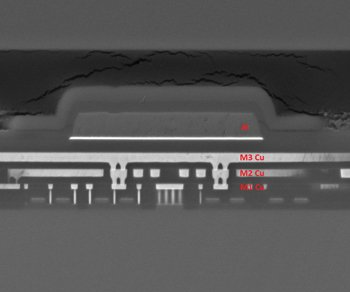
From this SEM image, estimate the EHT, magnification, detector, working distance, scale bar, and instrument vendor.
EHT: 10 kV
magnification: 20 K X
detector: BSED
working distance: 9.7 mm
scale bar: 5000 nm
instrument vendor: FEI